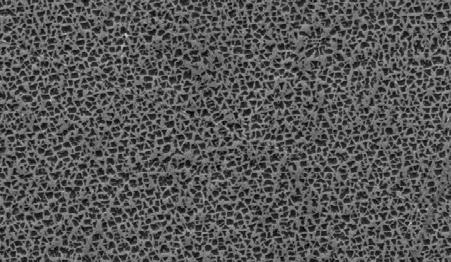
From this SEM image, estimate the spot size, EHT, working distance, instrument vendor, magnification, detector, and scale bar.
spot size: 4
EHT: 20 kV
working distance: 8.3 mm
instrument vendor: FEI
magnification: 2.5 K X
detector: SE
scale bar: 10000 nm